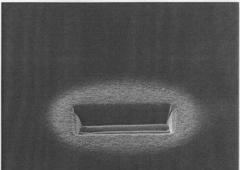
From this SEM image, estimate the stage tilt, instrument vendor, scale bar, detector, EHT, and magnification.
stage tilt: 45°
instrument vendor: FEI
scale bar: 5000 nm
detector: CDM-E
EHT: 30 kV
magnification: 12 K X